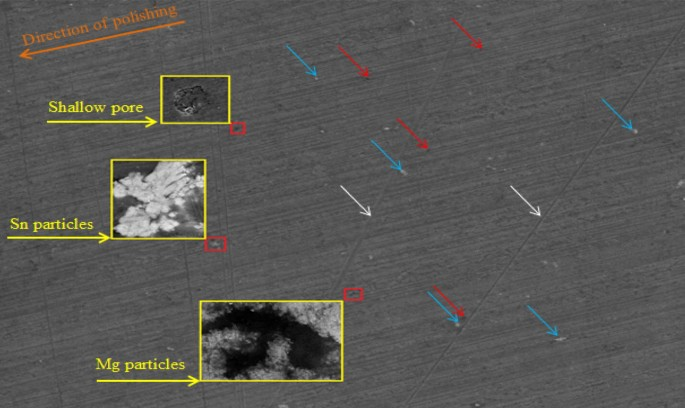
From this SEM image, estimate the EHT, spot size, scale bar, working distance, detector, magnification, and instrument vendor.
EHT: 20 kV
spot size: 3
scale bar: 100000 nm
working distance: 8.9 mm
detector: ETD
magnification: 1 K X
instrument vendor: FEI